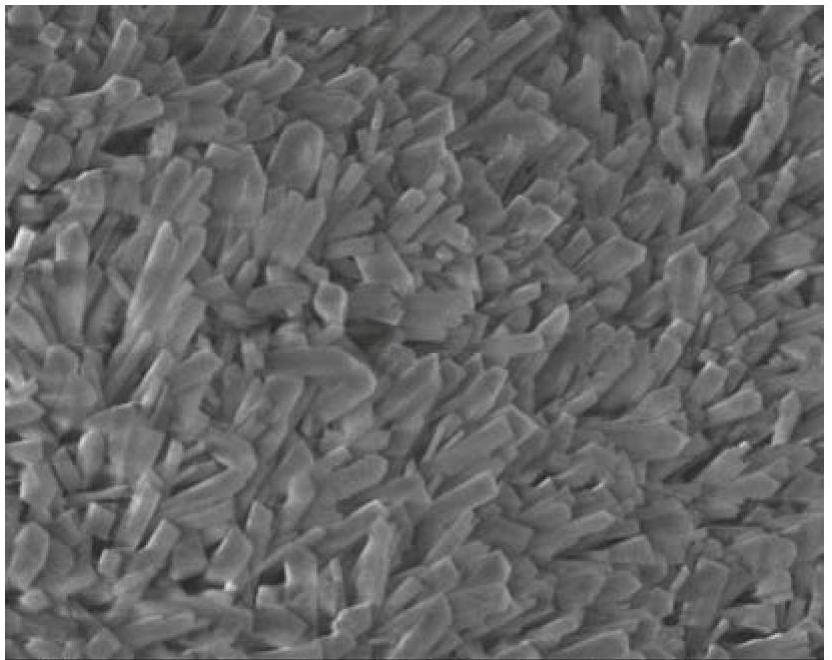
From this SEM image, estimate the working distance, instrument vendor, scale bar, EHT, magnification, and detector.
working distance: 8.5 mm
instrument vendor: JEOL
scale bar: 1000 nm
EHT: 5 kV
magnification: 10 K X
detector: SEI